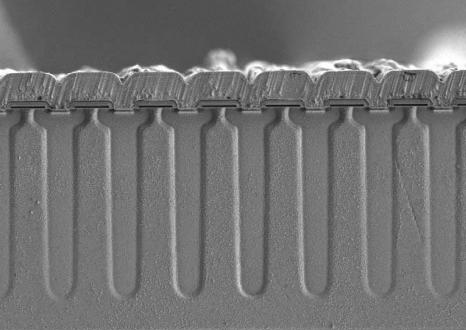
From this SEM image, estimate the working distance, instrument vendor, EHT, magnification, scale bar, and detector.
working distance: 10.2 mm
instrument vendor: Hitachi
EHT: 1 kV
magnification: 1.3 K X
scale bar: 40000 nm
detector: SE(L)